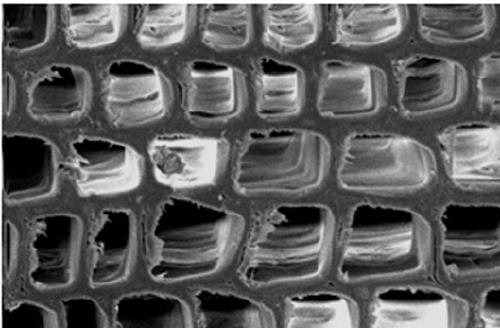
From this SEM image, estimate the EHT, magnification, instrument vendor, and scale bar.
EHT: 10 kV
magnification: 0.65 K X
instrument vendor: FEI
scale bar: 50000 nm